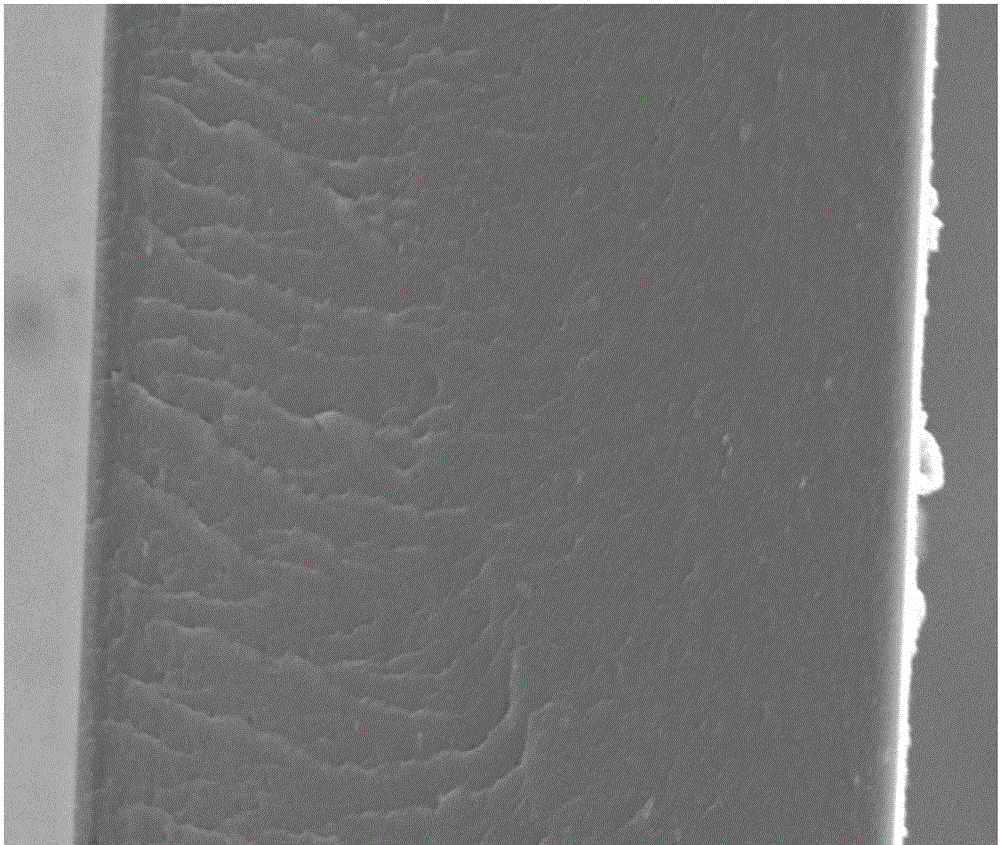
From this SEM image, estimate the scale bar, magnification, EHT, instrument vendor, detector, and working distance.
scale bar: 20000 nm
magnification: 5 K X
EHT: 10 kV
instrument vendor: FEI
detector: ETD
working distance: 12.2 mm